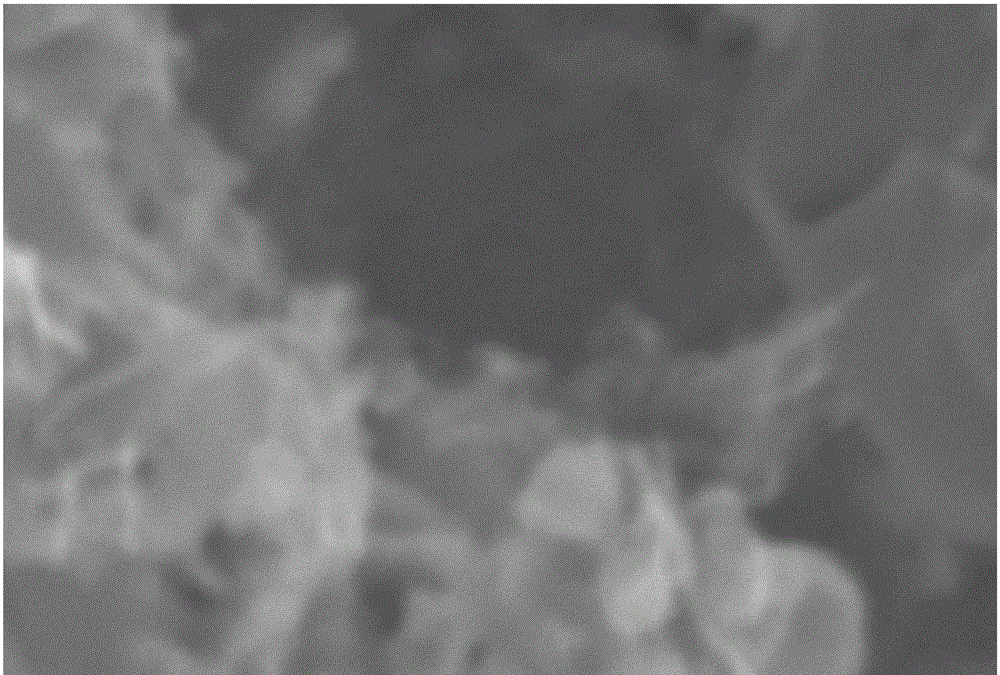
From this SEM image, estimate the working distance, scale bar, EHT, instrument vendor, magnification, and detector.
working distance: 23 mm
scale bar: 1000 nm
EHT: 15 kV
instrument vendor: JEOL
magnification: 15 K X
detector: SEI